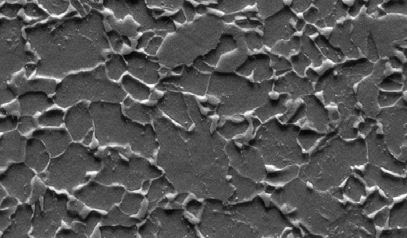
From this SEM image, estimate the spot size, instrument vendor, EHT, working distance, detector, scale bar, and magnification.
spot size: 4.5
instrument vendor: FEI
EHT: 30 kV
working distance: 10.1 mm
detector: SE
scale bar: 20000 nm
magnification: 1 K X